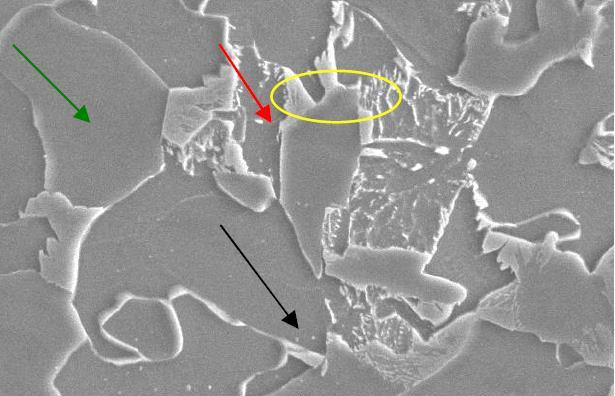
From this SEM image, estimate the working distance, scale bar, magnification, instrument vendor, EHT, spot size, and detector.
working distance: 10.2 mm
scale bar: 10000 nm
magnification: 6.5 K X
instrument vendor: FEI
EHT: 20 kV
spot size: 4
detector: SE